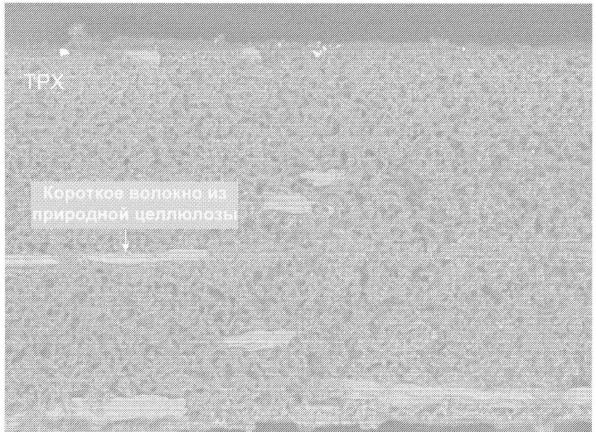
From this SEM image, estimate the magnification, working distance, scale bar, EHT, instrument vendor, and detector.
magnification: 0.4 K X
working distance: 16 mm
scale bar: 10000 nm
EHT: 10 kV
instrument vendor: JEOL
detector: COMPO